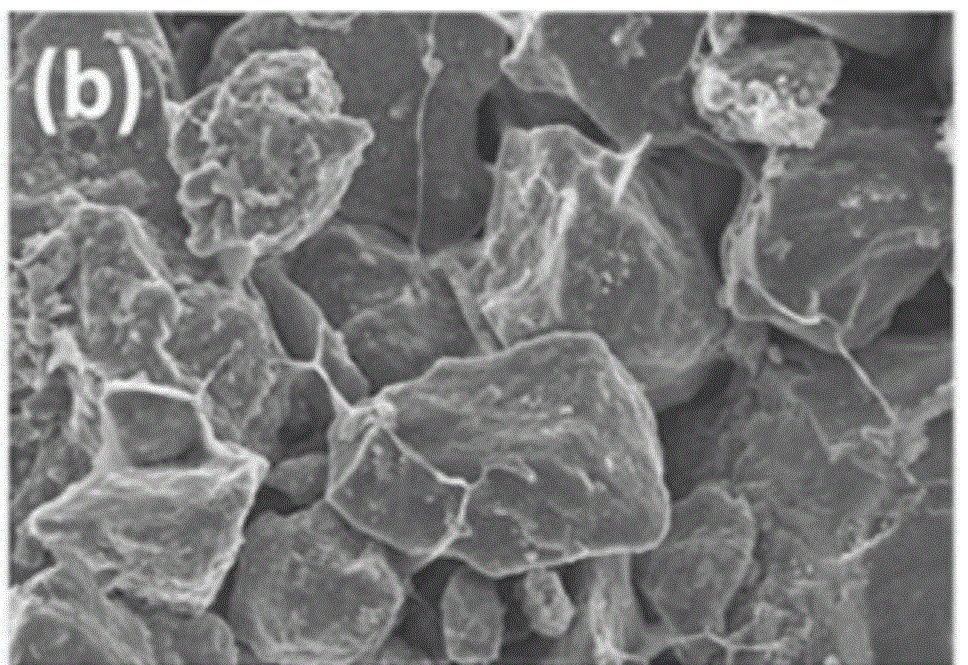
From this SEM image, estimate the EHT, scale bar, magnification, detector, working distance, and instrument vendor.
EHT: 20 kV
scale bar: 10000 nm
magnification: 0.5 K X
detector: InLens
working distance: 5 mm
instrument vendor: Zeiss